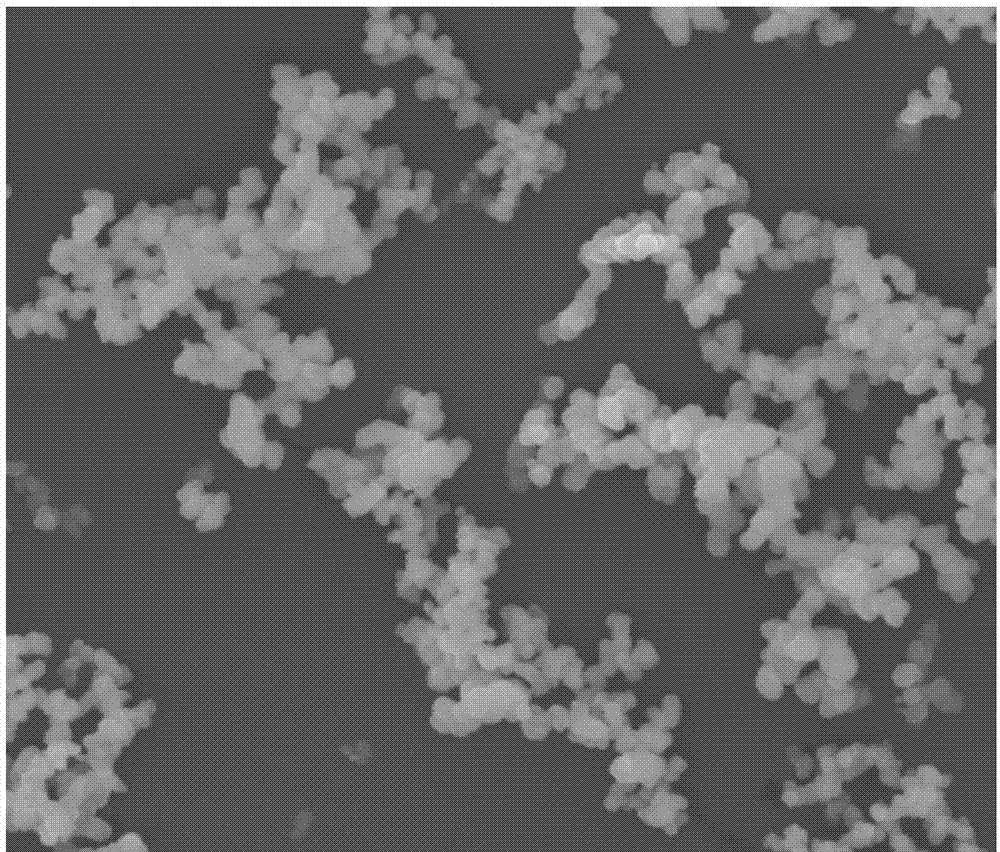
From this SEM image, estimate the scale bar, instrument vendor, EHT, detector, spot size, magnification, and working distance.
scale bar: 10000 nm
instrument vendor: FEI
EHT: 20 kV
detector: ETD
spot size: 4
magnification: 5 K X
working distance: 10.4 mm